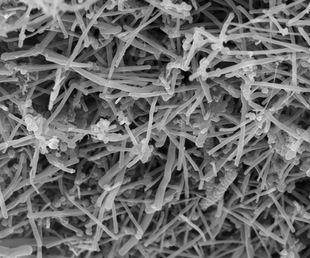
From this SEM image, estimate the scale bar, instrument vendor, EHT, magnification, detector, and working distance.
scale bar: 4000 nm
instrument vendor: FEI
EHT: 10 kV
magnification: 20 K X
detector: TLD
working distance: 4.8 mm